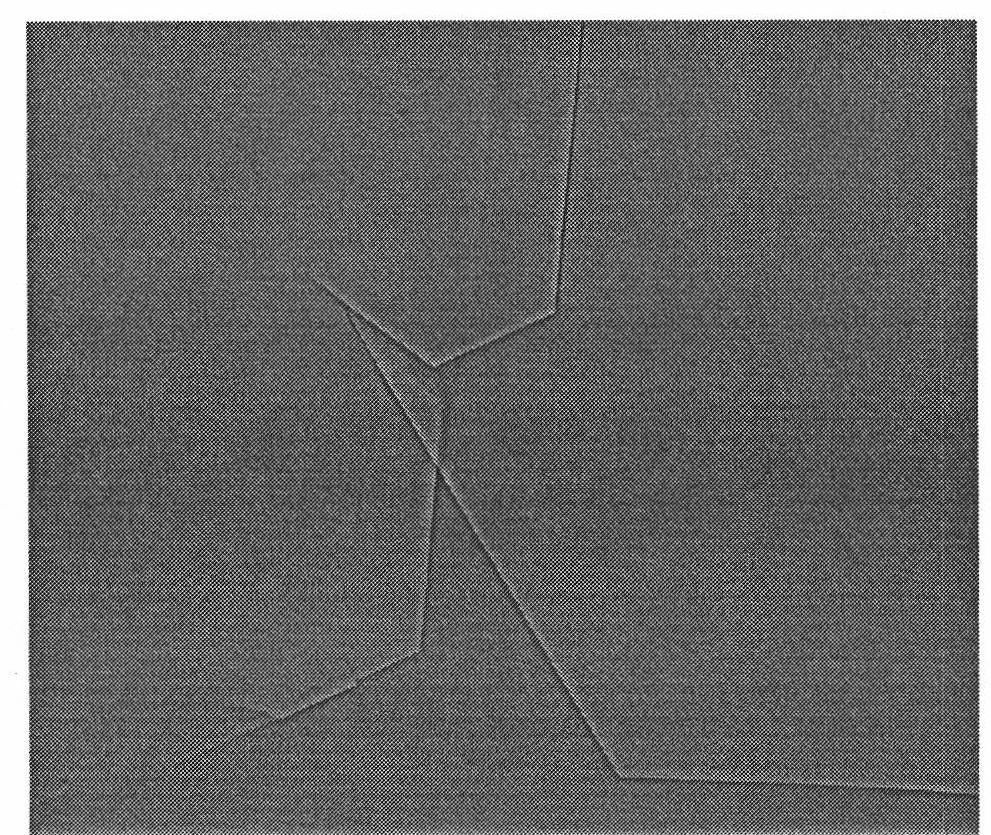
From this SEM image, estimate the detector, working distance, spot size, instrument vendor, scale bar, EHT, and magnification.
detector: SSD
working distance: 12.3 mm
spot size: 3.5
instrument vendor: FEI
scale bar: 100000 nm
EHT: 20 kV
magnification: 0.8 K X